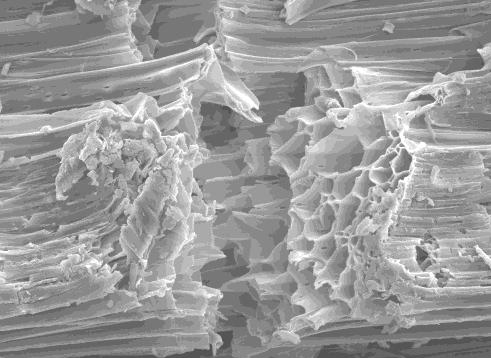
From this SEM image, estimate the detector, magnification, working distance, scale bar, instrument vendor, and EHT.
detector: SEI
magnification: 0.5 K X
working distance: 20 mm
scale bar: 10000 nm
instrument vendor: JEOL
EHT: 15 kV